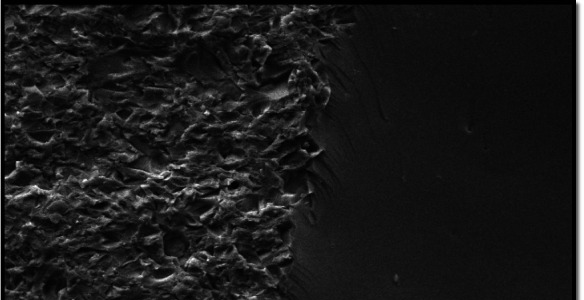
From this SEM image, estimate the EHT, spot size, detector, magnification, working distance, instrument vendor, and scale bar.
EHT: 20 kV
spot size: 4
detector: SE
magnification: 1 K X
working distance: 11.3 mm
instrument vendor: FEI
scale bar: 50000 nm